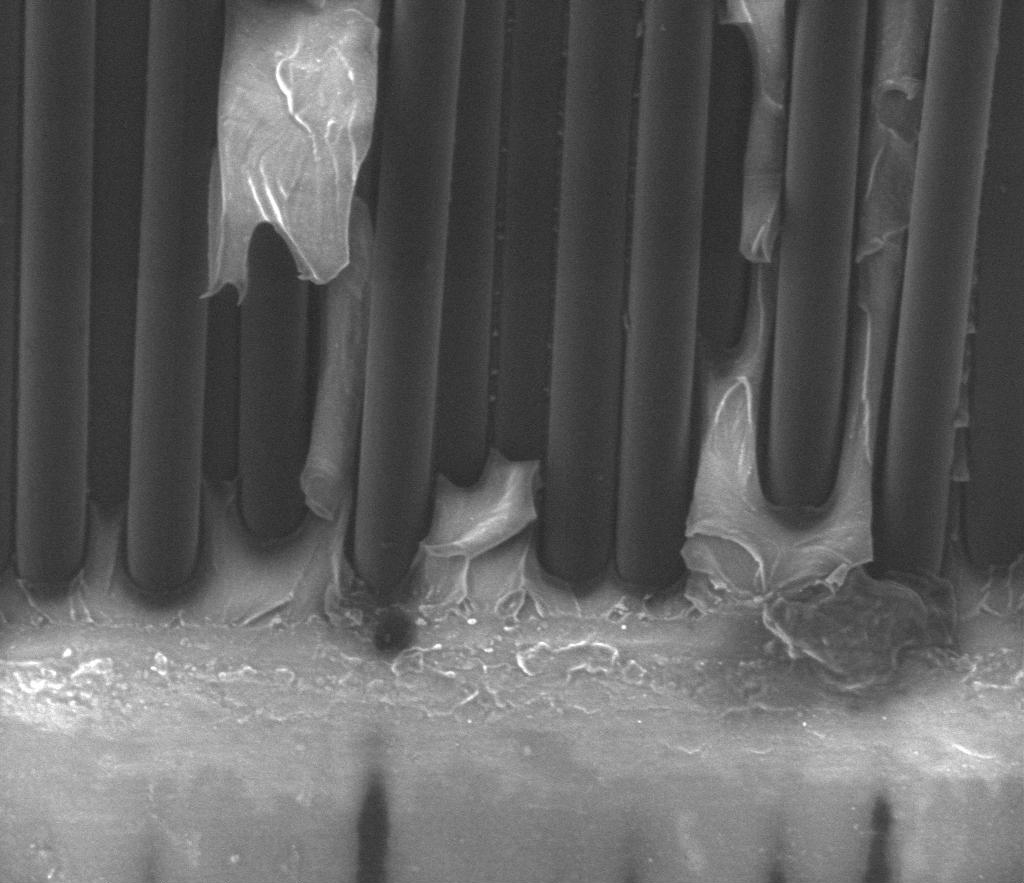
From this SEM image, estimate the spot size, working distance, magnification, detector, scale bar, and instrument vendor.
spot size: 4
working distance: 11 mm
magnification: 2.5 K X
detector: SE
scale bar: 50000 nm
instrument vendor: FEI